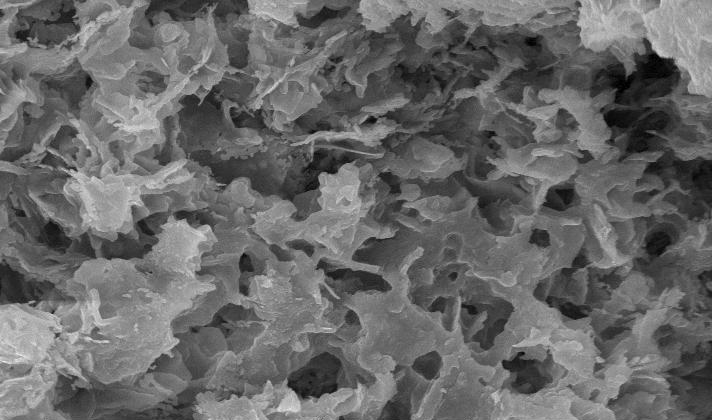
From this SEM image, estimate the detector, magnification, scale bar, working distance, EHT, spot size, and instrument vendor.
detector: SE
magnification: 2.5 K X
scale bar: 10000 nm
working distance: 12 mm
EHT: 20 kV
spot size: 3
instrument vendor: FEI